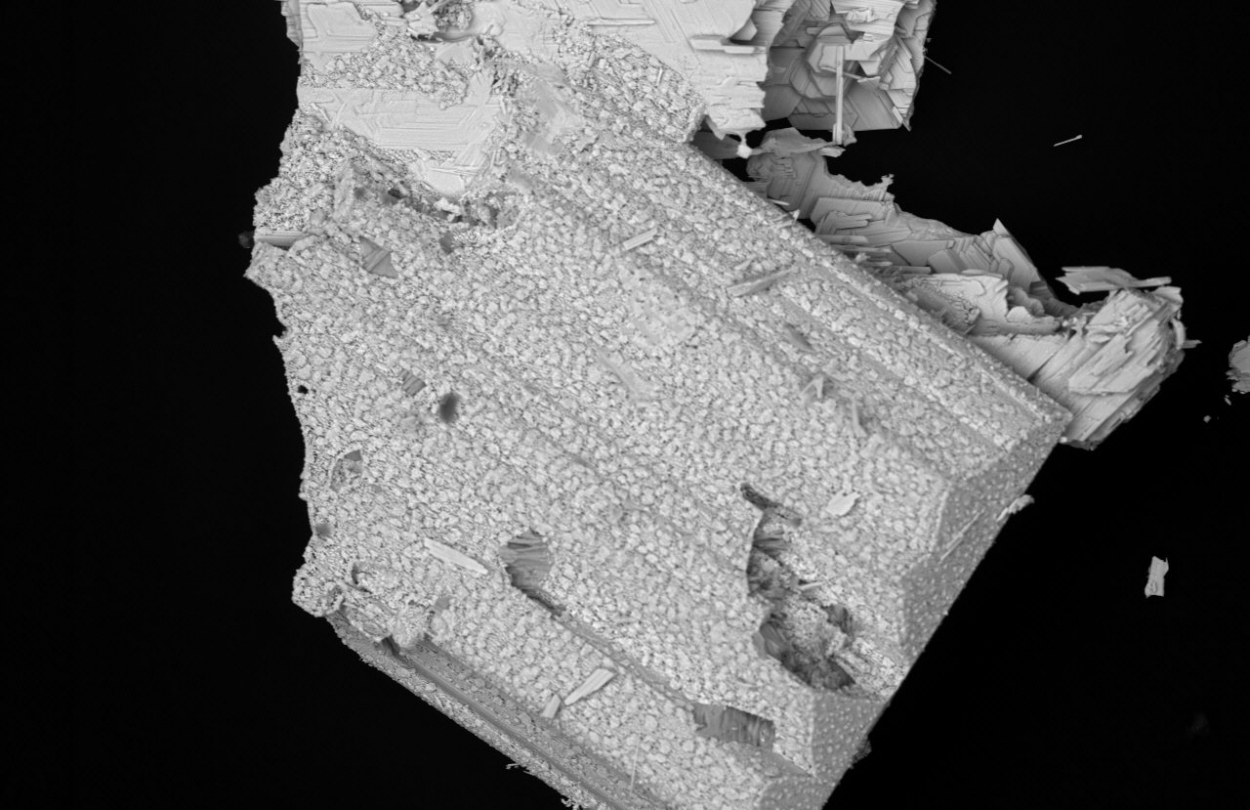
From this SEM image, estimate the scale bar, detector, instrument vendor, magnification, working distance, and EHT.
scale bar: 100000 nm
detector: BSE3D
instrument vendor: Hitachi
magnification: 0.3 K X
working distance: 11.3 mm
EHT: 20 kV